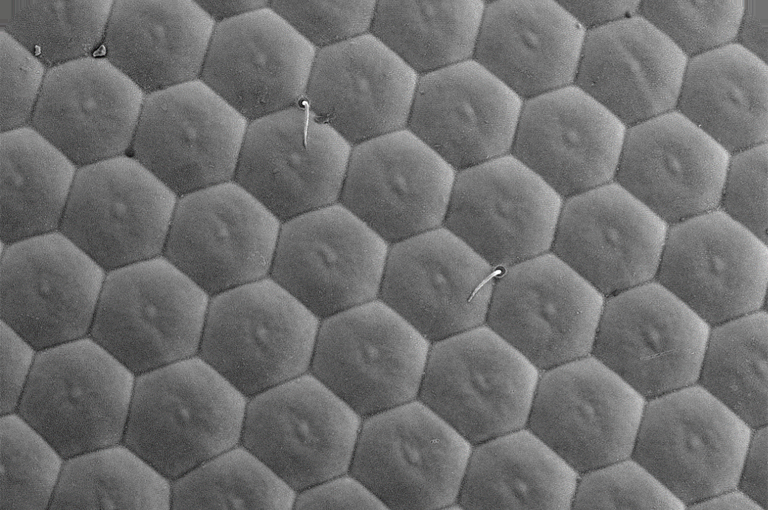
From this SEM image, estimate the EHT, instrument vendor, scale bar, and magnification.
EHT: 10 kV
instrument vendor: JEOL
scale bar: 20000 nm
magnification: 0.85 K X